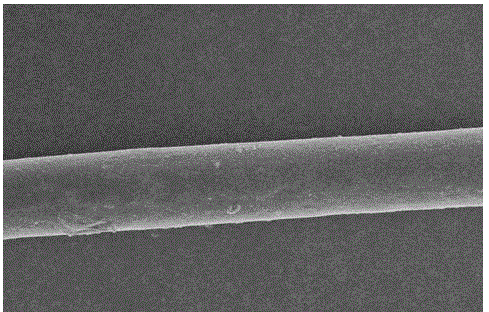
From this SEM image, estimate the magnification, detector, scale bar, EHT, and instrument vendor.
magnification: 0.5 K X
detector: SE(U)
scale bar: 100000 nm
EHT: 10 kV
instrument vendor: Hitachi